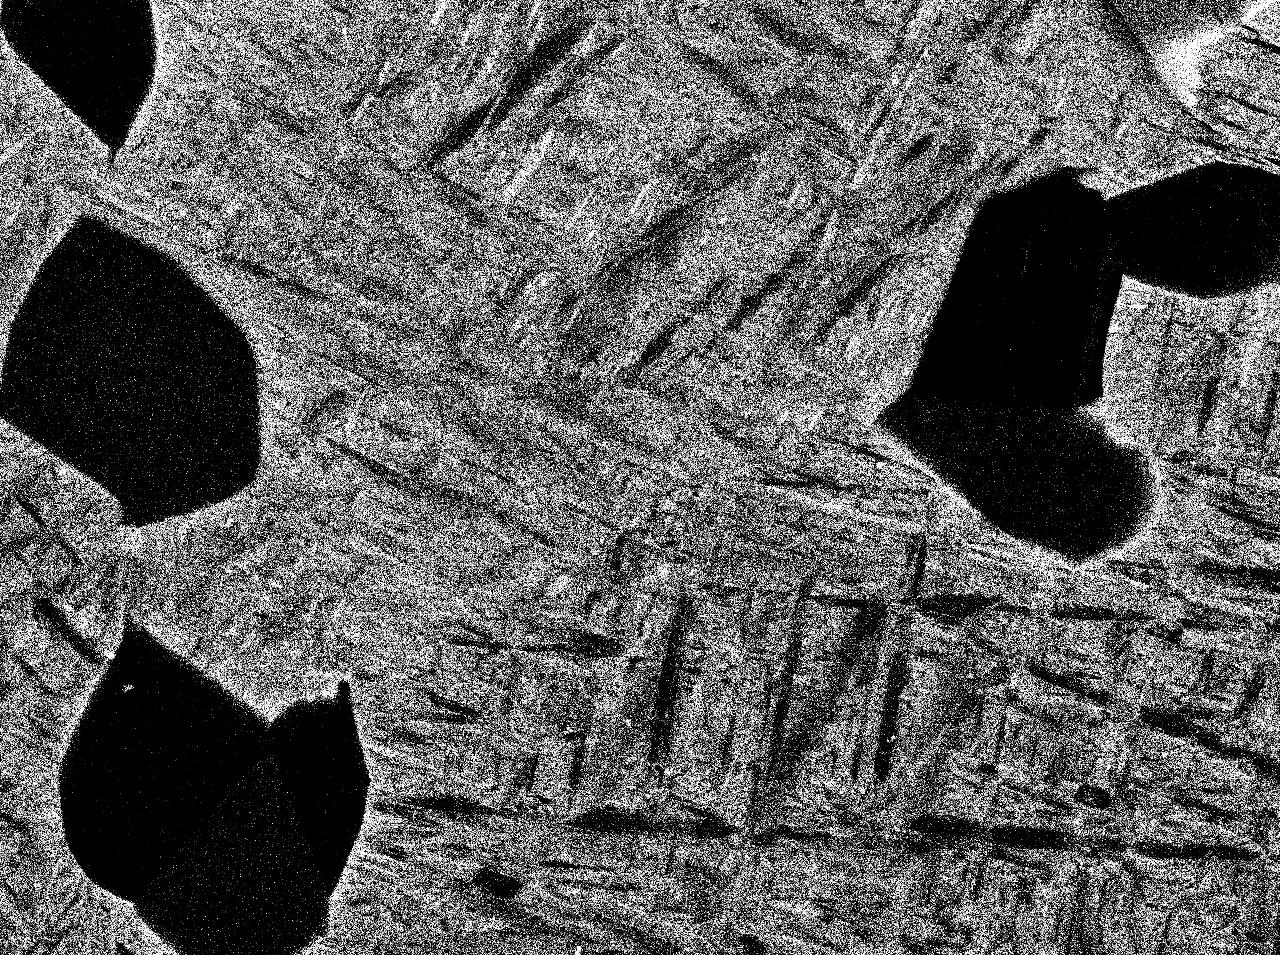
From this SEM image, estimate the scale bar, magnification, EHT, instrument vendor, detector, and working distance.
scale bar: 1000 nm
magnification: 5 K X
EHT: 15 kV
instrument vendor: JEOL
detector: COMPO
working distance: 10 mm